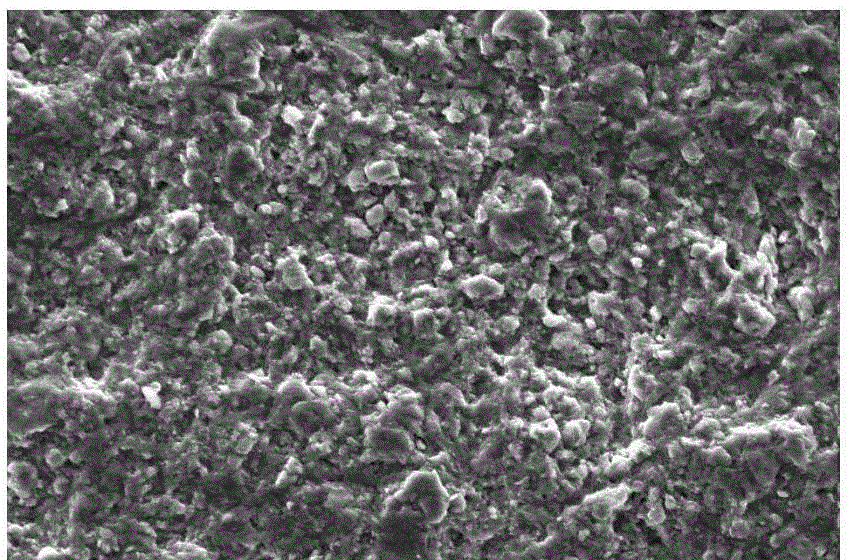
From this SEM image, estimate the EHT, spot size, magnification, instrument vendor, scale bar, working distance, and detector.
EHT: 20 kV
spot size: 4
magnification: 1 K X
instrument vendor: FEI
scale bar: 50000 nm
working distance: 9.9 mm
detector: SE